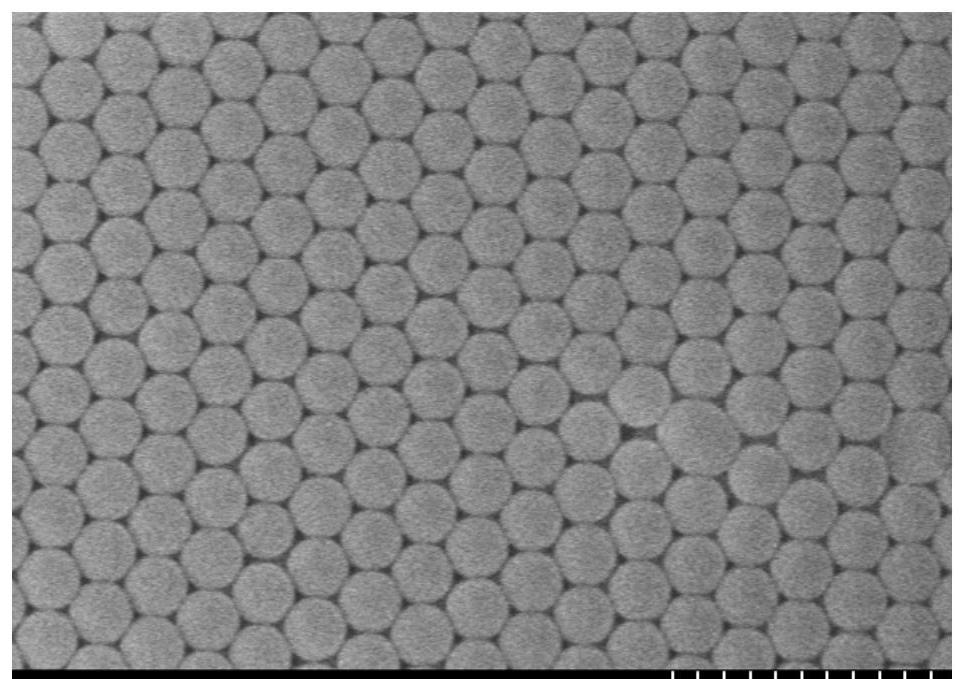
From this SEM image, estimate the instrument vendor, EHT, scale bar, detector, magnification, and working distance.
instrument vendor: Hitachi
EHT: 5 kV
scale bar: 1000 nm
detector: SE(U)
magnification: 35 K X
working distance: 13.6 mm